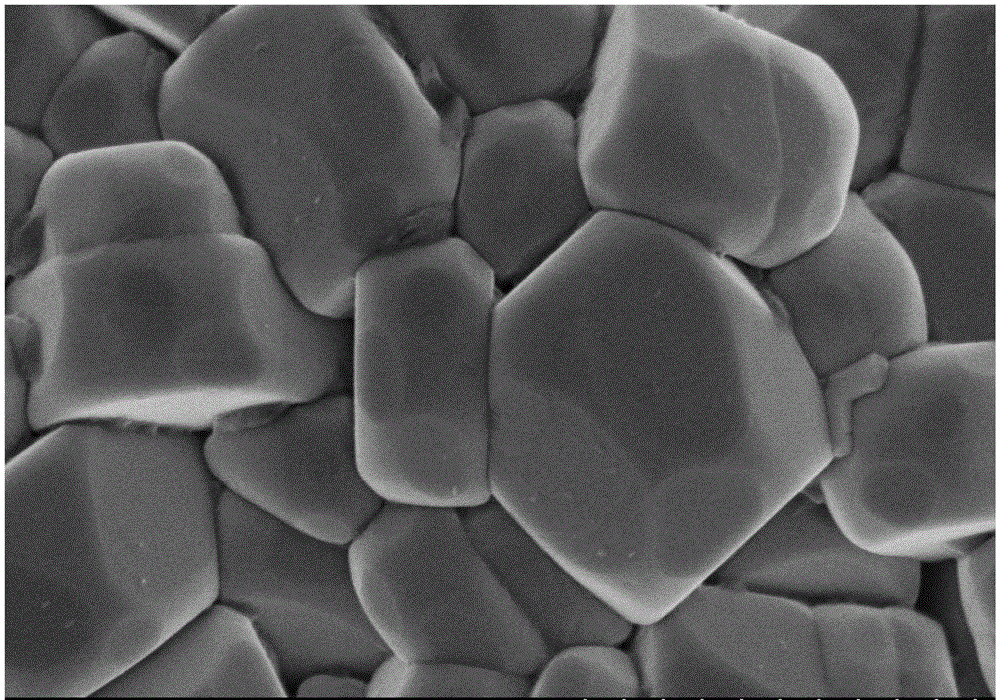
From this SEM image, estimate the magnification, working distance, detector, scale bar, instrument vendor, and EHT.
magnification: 50 K X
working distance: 8 mm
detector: SE(M)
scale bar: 1000 nm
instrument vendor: Hitachi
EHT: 3 kV